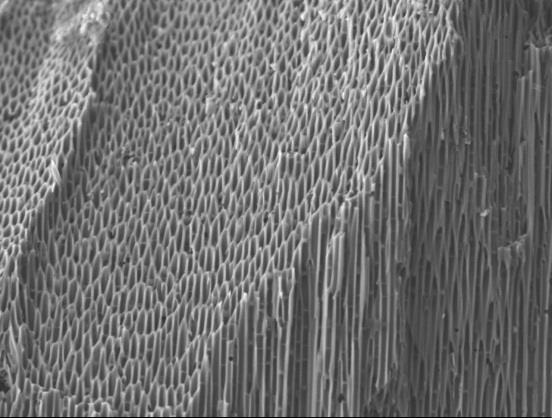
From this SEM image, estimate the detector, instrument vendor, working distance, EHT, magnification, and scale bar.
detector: LEI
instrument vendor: JEOL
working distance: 8.2 mm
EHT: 5 kV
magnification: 4.5 K X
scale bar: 1000 nm